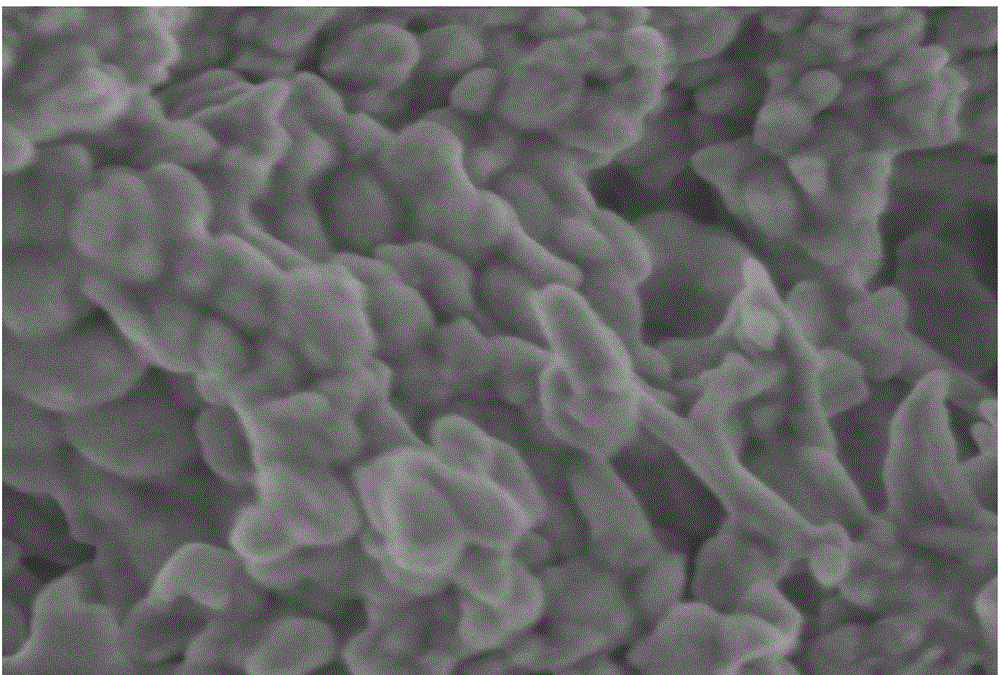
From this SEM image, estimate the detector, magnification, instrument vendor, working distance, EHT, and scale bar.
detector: SE+BSE(TU)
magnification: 150 K X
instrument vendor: Hitachi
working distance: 2.5 mm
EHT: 1 kV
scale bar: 300 nm